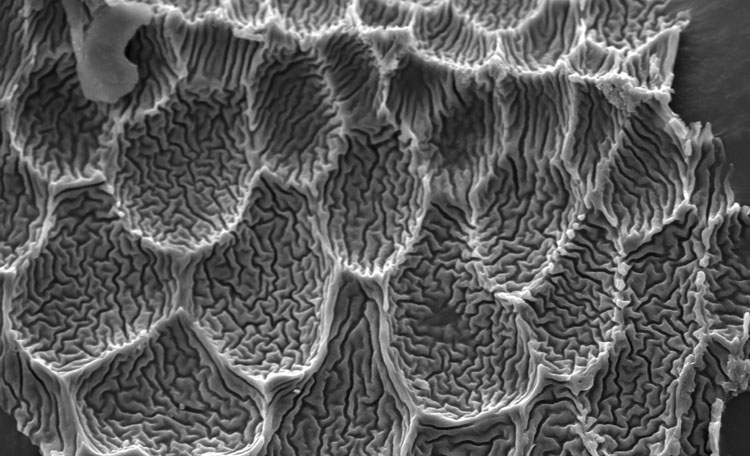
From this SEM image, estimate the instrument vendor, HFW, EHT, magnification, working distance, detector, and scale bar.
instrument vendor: Thermo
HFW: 96.6 µm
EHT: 10 kV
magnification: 5.4 K X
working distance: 5.947 mm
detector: SED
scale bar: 30000 nm